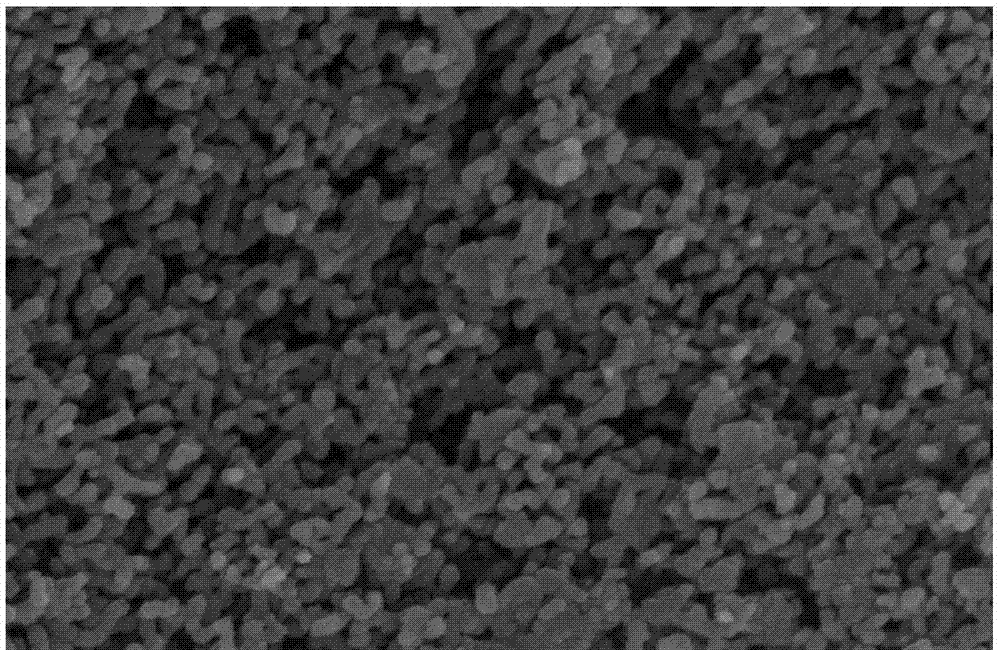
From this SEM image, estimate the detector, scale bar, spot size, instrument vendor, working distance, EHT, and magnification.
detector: TLD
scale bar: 500 nm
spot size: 3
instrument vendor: FEI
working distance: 6.1 mm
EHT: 20 kV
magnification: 100 K X